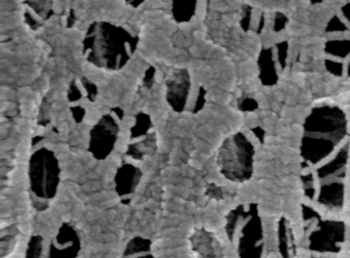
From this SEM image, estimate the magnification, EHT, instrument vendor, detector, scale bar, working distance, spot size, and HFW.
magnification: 100 K X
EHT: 5 kV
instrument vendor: FEI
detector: TLD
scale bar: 200 nm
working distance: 5.6 mm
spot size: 3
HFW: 1.2 µm